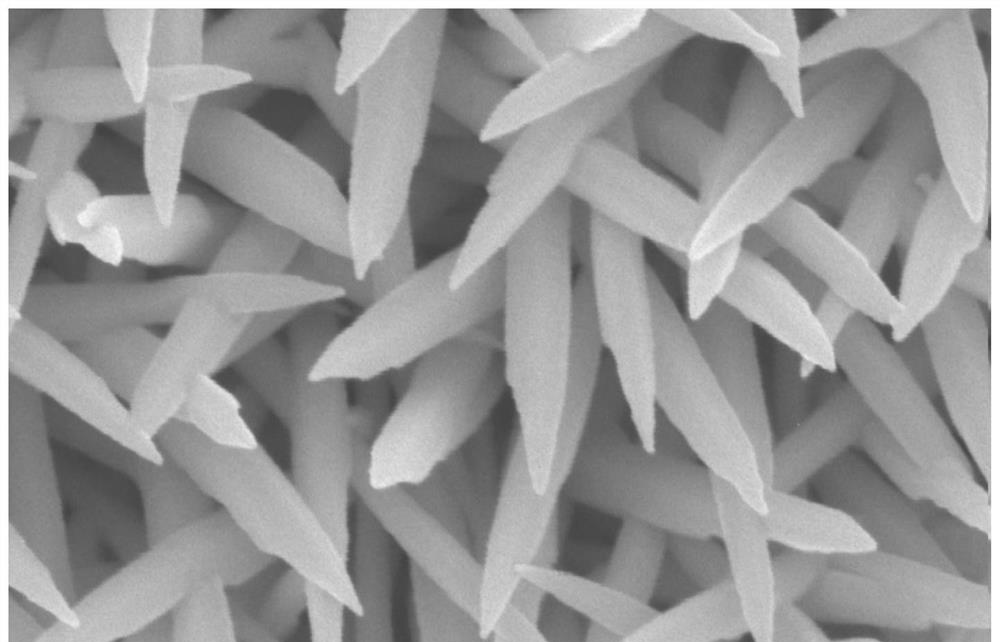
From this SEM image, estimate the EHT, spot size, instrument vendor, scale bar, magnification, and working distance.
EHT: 10 kV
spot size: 4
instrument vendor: FEI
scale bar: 500 nm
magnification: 50 K X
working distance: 6.3 mm